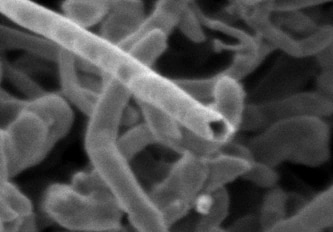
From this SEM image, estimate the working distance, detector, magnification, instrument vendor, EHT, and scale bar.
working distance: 2.9 mm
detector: SE(T)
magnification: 200 K X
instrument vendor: Hitachi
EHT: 0.5 kV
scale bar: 200 nm